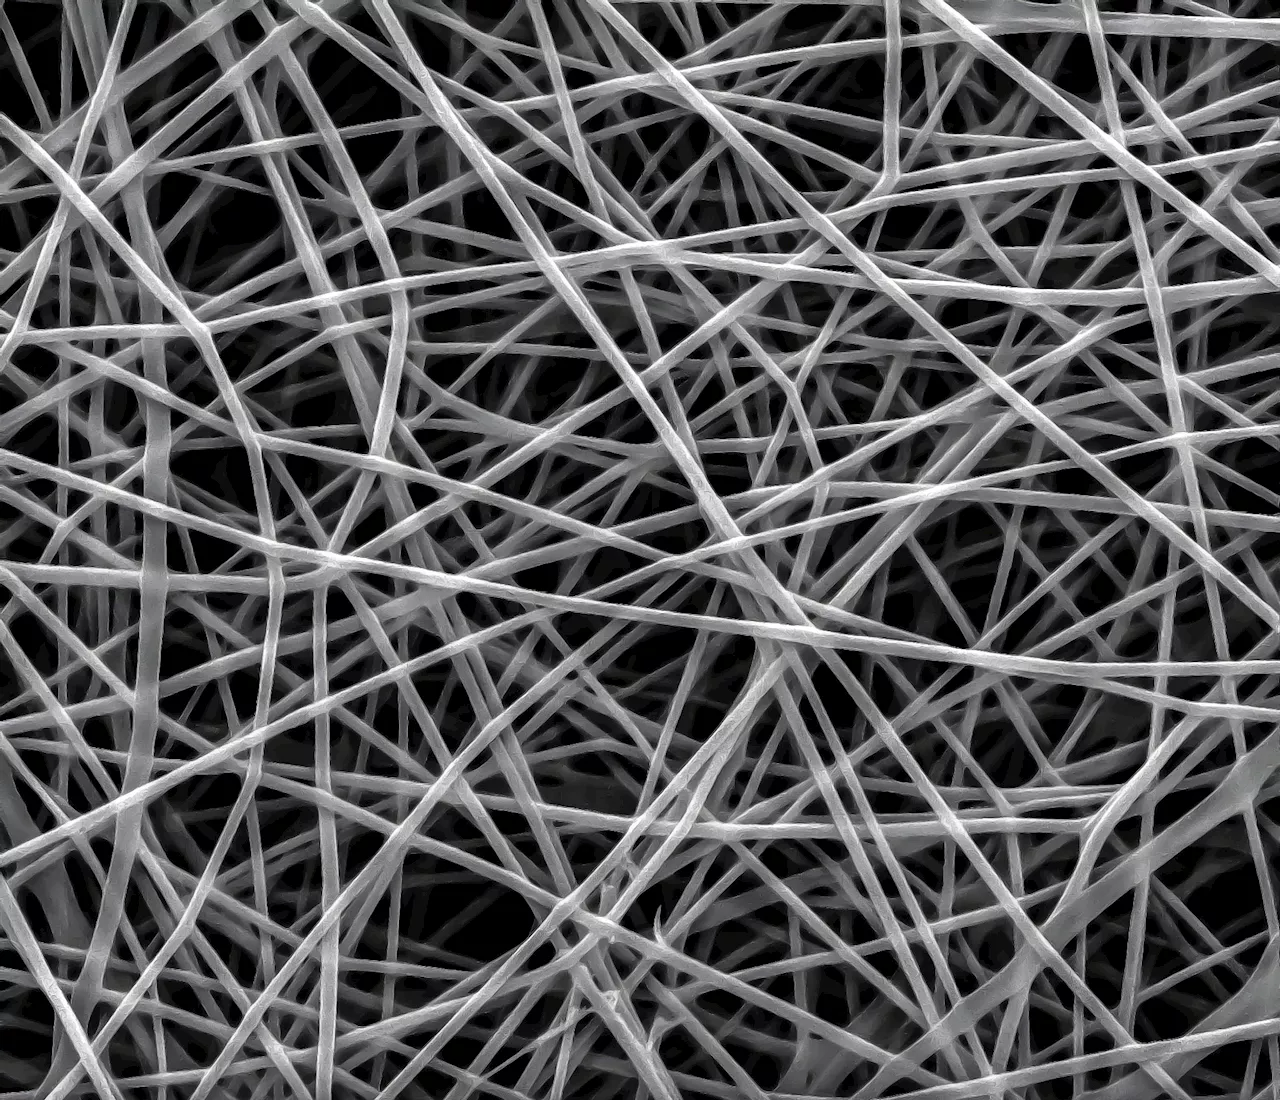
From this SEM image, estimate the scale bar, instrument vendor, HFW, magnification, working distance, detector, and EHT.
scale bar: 20000 nm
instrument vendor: FEI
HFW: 54.08 µm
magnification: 5 K X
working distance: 8.9 mm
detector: SE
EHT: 25 kV